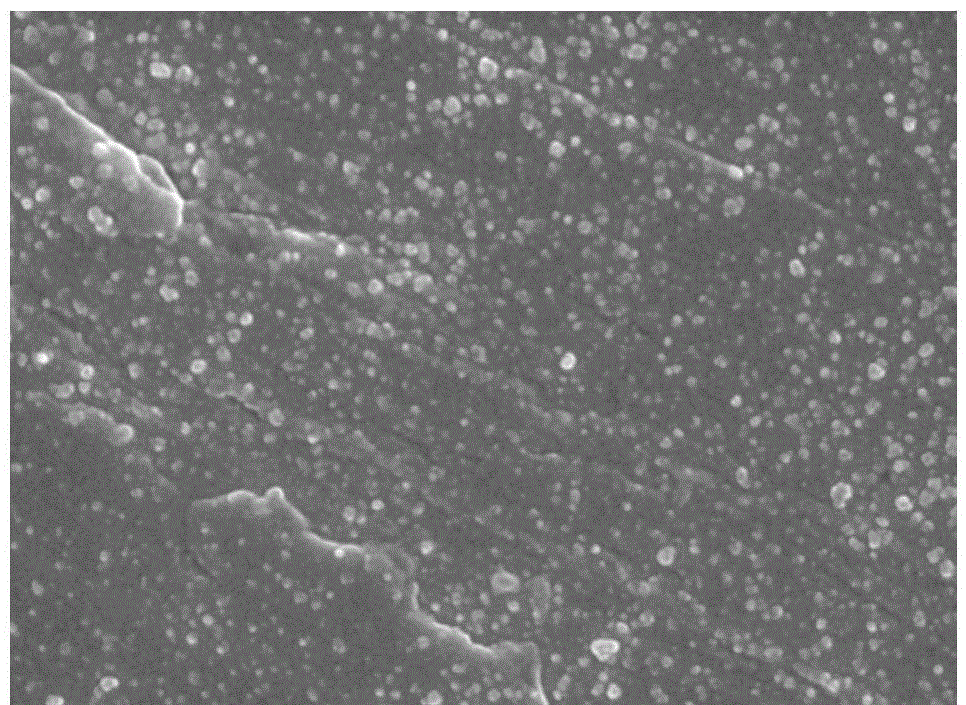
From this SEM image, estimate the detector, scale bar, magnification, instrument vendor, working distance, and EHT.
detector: SEI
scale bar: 100 nm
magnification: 50 K X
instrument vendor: JEOL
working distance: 7.5 mm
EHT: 5 kV